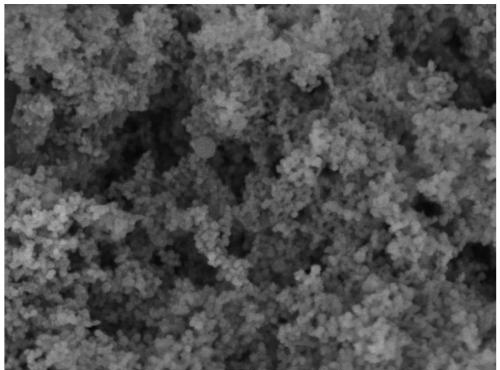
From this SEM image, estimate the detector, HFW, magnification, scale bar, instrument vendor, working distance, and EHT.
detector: In-Beam SE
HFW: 2.77 µm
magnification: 100 K X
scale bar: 500 nm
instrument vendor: Tescan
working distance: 4 mm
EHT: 5 kV